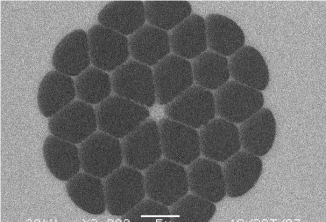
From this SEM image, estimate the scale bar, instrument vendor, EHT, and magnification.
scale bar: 5000 nm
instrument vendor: JEOL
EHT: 20 kV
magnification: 3 K X